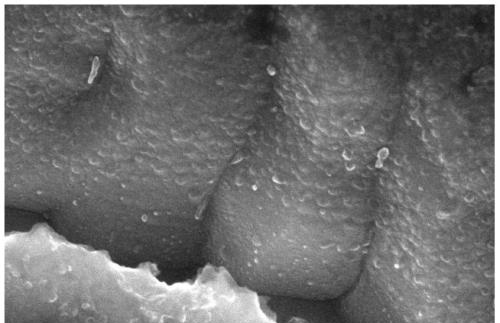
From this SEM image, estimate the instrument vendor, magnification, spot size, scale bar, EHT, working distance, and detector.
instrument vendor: FEI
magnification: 80 K X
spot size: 3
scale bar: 500 nm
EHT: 15 kV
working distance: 5.2 mm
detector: TLD